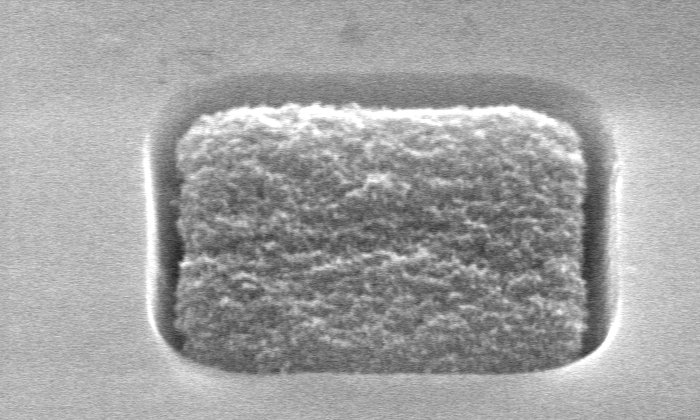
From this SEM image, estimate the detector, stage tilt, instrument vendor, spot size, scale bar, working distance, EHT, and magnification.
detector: SE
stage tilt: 45°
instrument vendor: FEI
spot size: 2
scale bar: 500 nm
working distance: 9.9 mm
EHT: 15 kV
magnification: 80 K X